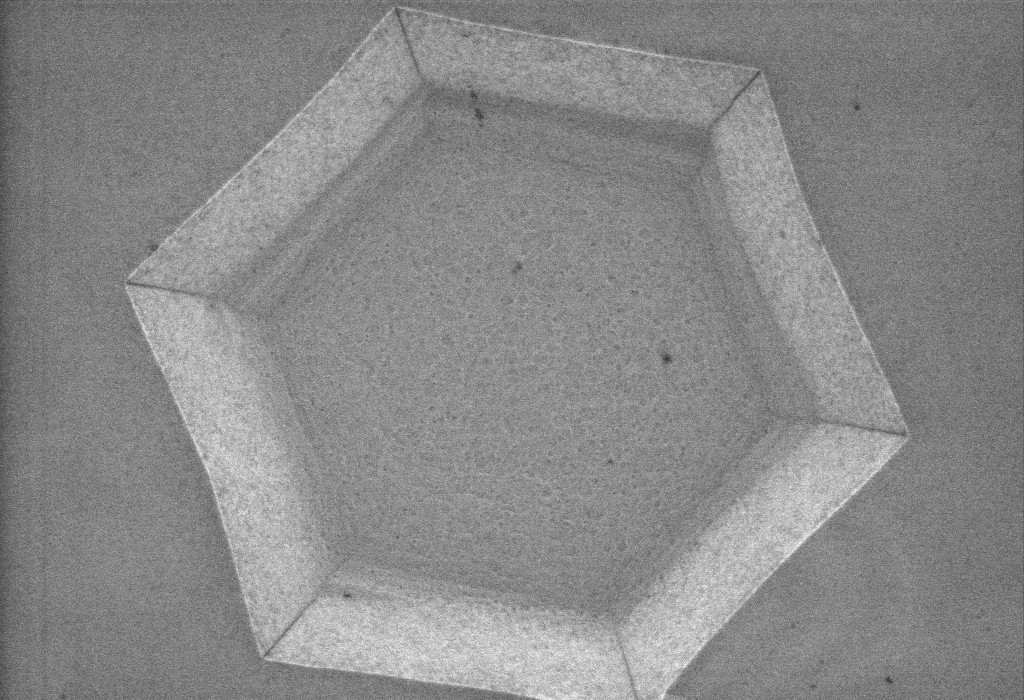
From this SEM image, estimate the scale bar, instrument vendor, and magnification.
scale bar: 200 nm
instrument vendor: Zeiss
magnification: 25 K X